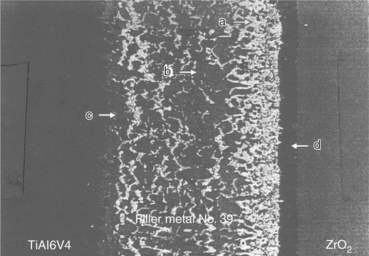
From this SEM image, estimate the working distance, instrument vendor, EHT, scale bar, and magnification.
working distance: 12 mm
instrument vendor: Zeiss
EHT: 20 kV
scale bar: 50000 nm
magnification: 0.5 K X